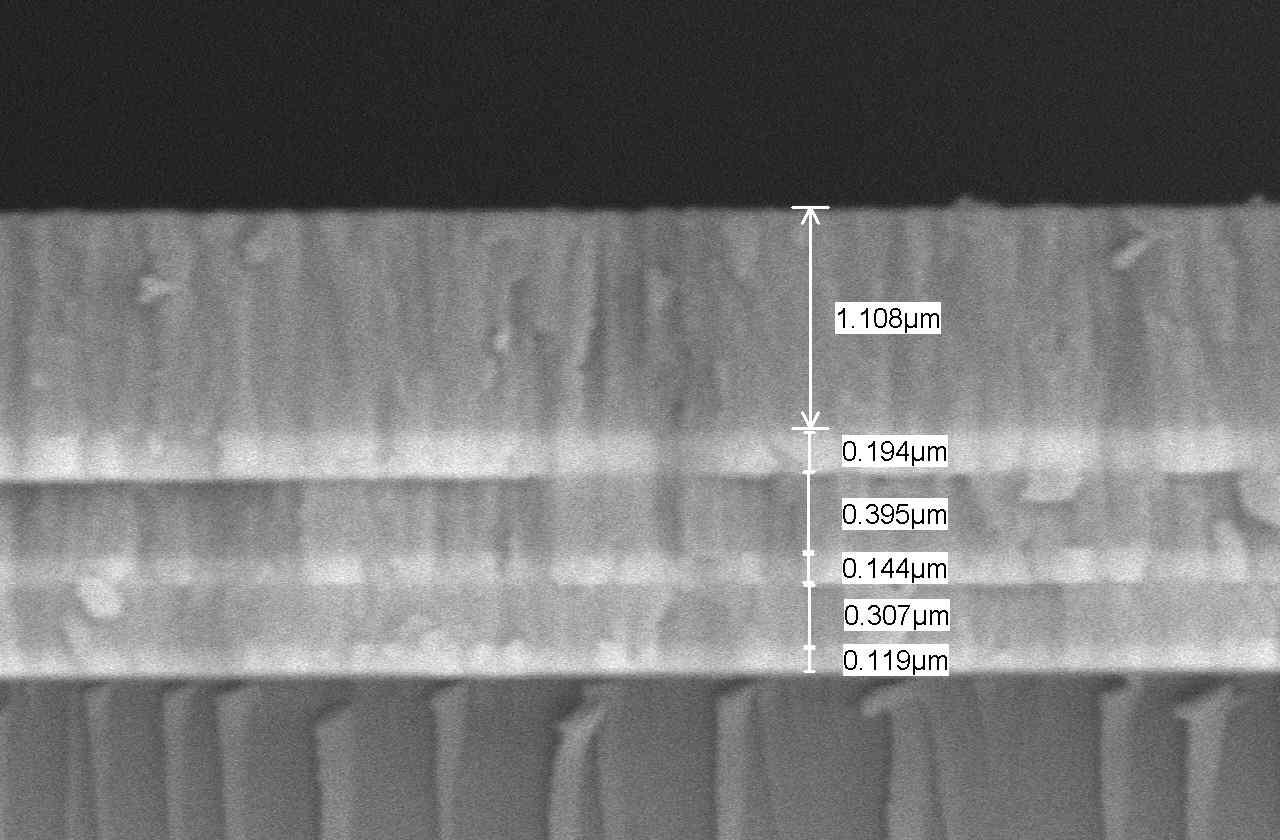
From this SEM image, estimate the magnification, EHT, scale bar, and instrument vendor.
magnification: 20 K X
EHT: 20 kV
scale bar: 1000 nm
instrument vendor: JEOL